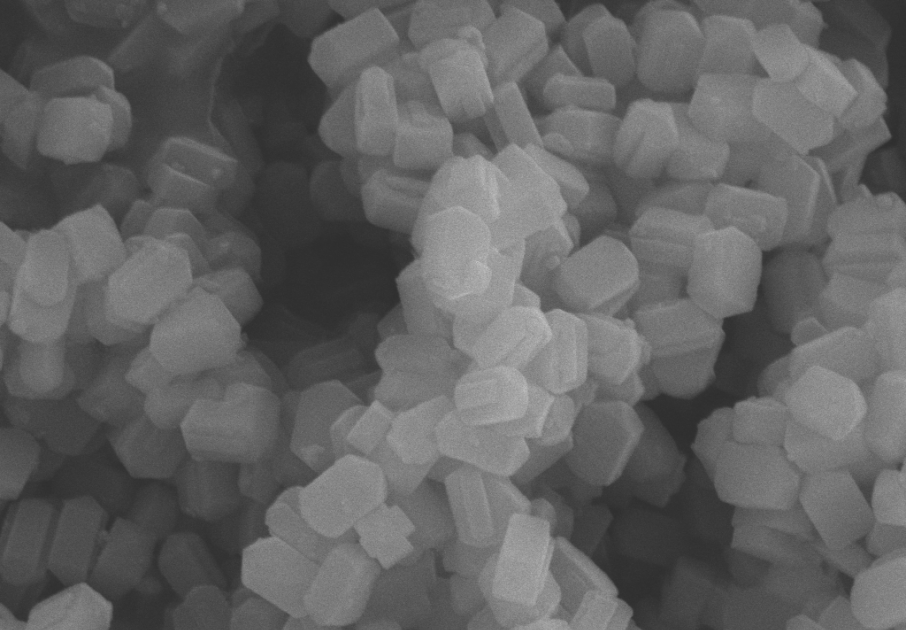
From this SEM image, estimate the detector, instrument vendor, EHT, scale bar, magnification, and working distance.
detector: SE2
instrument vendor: Zeiss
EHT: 10 kV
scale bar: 200 nm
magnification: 20 K X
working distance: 10.5 mm